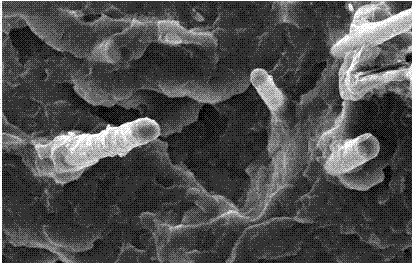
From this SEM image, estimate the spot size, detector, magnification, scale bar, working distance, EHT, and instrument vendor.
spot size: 3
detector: SE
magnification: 2 K X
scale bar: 20000 nm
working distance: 8.1 mm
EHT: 20 kV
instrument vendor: FEI